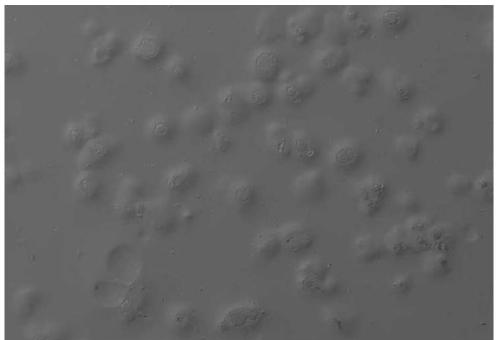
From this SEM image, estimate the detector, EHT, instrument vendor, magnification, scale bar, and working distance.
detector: BSE-TOPO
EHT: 15 kV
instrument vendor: Hitachi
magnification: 0.065 K X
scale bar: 500000 nm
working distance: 5.9 mm